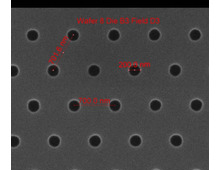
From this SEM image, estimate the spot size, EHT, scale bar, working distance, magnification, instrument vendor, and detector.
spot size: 1.5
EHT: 10 kV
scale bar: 1000 nm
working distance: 10.4 mm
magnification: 75 K X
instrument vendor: FEI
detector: ETD